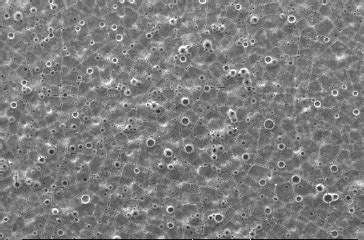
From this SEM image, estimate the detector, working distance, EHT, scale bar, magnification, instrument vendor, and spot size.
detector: SE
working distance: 9.4 mm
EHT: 20 kV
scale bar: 100000 nm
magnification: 0.2 K X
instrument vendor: FEI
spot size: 5